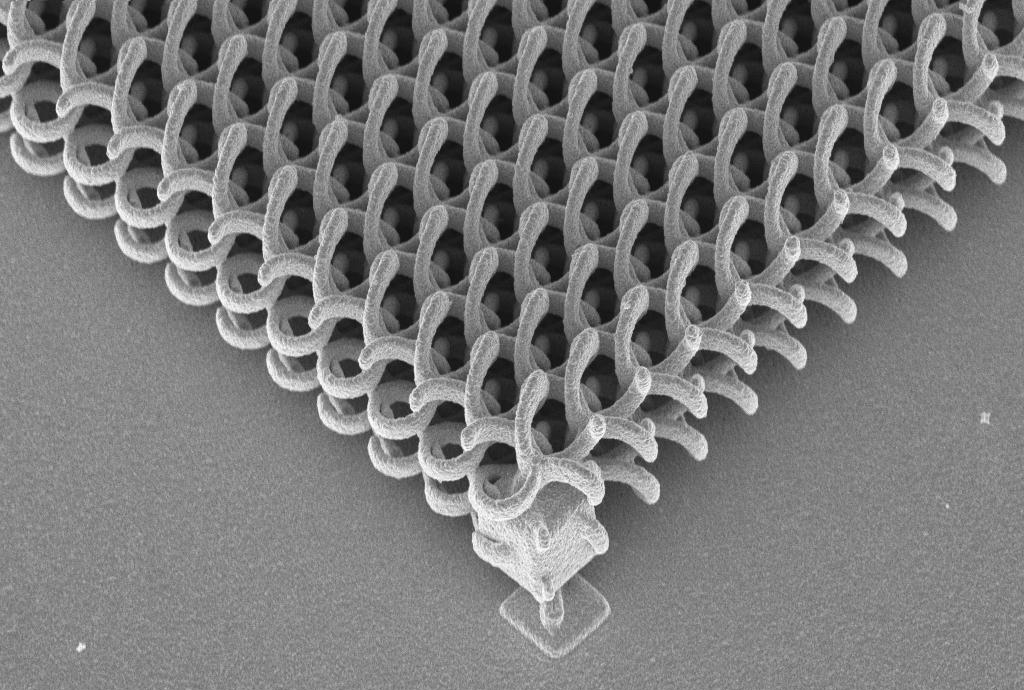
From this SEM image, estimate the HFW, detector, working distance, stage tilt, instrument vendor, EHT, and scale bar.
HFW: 40.81 µm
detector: InLens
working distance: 4 mm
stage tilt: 30°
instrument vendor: Zeiss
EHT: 5 kV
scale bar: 2000 nm